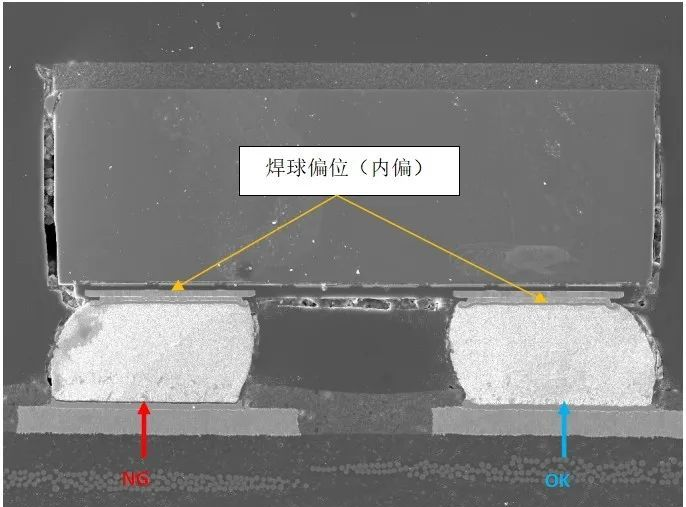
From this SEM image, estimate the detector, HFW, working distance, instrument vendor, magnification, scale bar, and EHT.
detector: SE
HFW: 652 µm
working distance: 29.99 mm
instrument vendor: Tescan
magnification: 0.425 K X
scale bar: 200000 nm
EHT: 20 kV